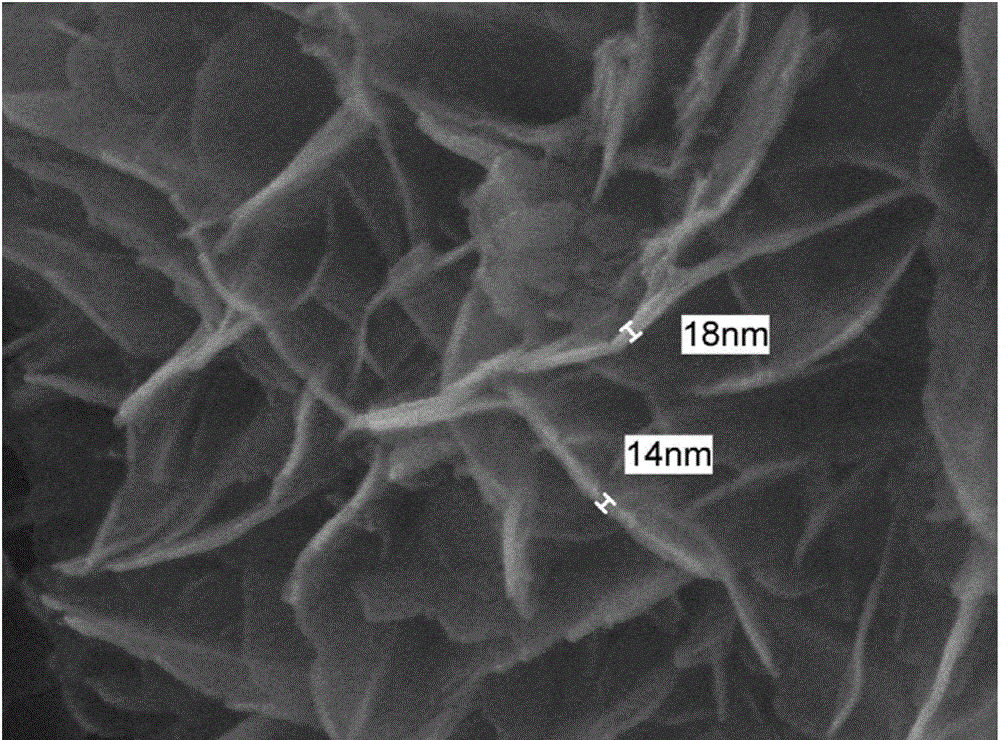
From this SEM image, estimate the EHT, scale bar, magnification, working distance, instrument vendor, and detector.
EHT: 15 kV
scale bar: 100 nm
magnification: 100 K X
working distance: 5.9 mm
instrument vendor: JEOL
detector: SEI